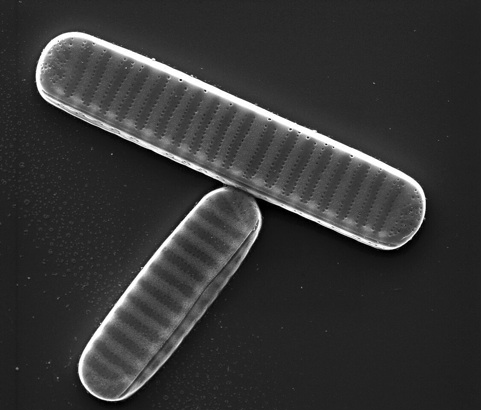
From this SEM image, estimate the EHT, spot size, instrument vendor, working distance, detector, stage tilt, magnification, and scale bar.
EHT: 10 kV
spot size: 3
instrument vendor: FEI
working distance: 9.5 mm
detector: SE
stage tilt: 0.7°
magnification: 15 K X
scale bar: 5000 nm